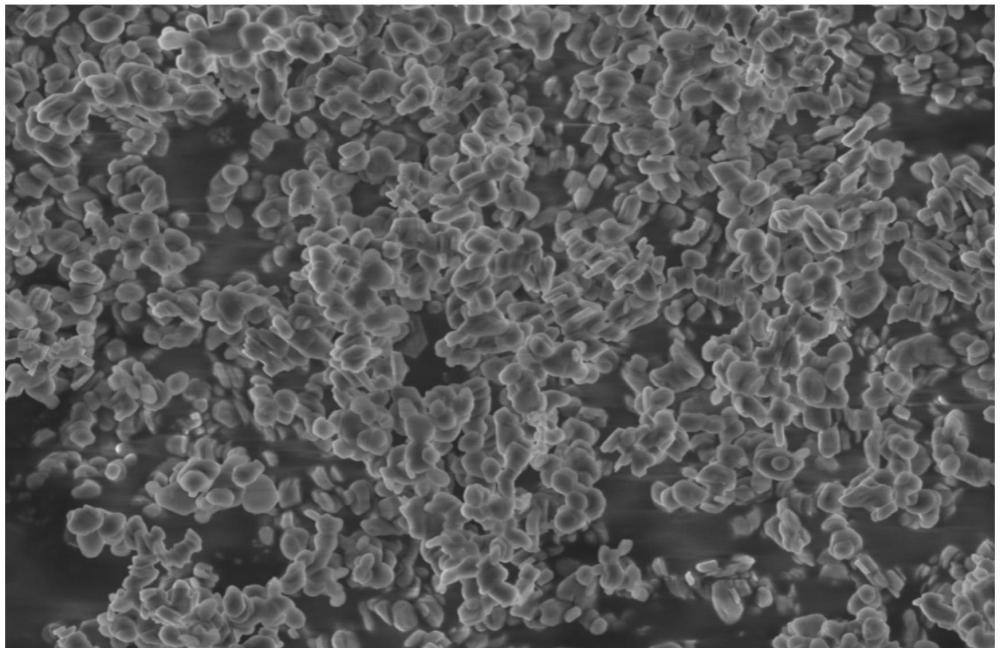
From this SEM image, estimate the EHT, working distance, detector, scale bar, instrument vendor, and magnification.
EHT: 3 kV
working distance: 8 mm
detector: SE(U)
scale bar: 5000 nm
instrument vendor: Hitachi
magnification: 10 K X